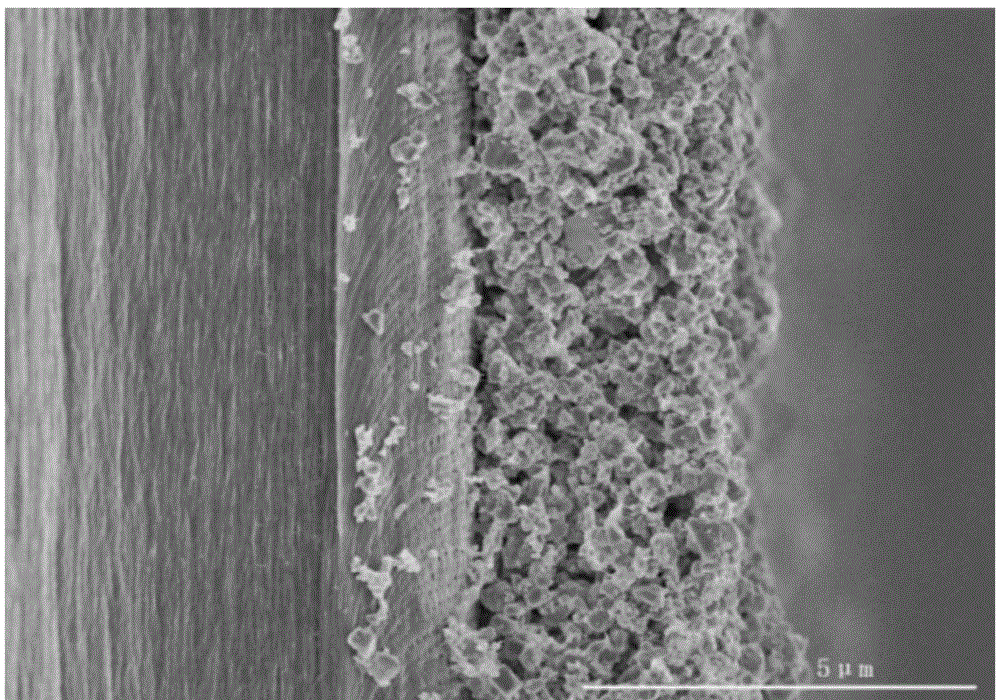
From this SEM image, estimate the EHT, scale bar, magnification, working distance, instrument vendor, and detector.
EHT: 4 kV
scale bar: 5000 nm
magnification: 10 K X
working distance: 7.6 mm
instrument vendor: Hitachi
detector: SE(M)